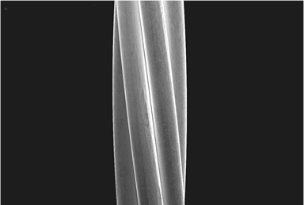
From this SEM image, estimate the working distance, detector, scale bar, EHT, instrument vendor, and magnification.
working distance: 7.8 mm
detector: SE(M)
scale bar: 50000 nm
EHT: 15 kV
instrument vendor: Hitachi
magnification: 1 K X